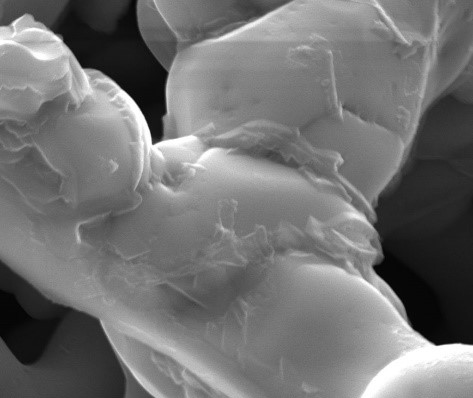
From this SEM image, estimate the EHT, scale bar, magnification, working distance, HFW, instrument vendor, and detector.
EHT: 20 kV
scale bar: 5000 nm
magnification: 10 K X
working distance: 10.3 mm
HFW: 12.7 µm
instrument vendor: FEI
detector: ETD